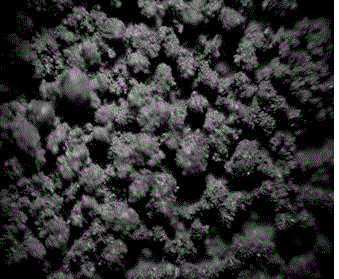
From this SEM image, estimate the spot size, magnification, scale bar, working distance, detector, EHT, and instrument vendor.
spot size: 3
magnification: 0.4 K X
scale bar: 200000 nm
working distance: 10 mm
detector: BSED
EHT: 30 kV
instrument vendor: FEI